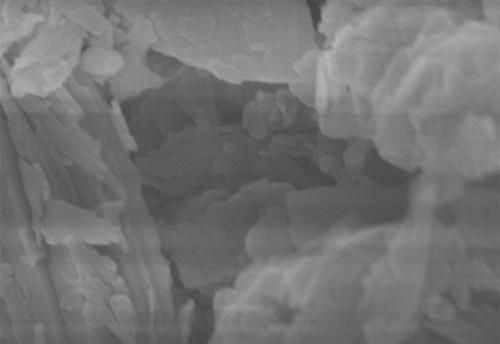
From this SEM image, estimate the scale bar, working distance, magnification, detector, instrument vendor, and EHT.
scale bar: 500 nm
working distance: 8.5 mm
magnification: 70 K X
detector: SE(UL)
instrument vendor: Hitachi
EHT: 5 kV